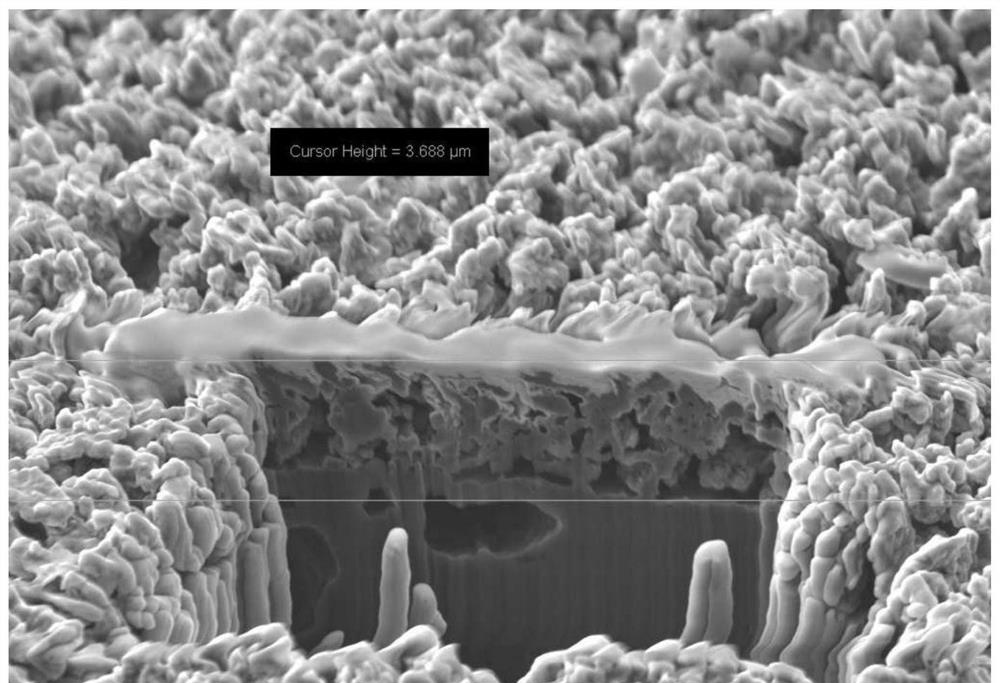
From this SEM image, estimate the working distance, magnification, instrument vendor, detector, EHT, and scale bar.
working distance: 4.9 mm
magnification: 4.42 K X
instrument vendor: Zeiss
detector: SE2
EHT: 5 kV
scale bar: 1000 nm